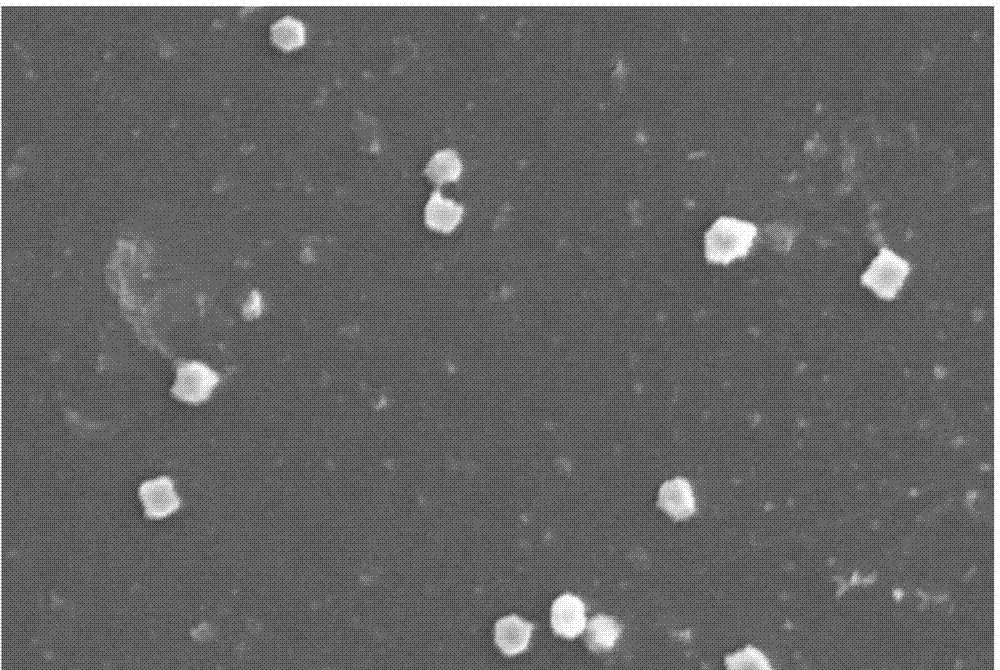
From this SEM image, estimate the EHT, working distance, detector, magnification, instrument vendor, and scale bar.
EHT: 10 kV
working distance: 15.3 mm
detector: SE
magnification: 3 K X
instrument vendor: Hitachi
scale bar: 10000 nm